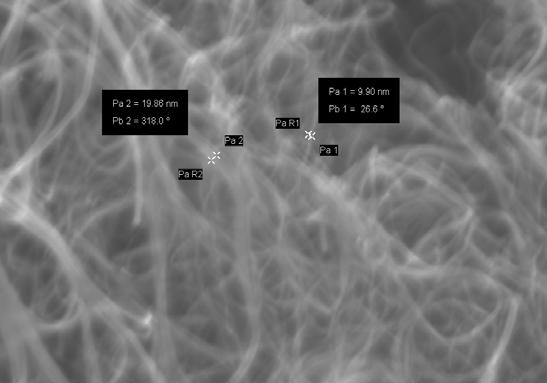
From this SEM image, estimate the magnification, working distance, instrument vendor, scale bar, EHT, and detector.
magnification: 75.65 K X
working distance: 4 mm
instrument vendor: Zeiss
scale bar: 200 nm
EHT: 10 kV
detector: InLens